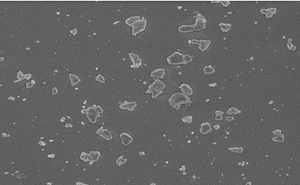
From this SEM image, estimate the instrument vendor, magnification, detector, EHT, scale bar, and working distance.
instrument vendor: JEOL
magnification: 3 K X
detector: SEI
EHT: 5 kV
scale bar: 1000 nm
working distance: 9.7 mm